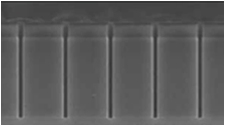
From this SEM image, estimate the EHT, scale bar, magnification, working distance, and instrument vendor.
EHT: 5 kV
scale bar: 2000 nm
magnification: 25 K X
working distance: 12 mm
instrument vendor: Hitachi